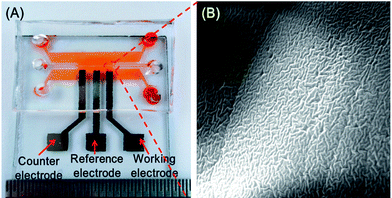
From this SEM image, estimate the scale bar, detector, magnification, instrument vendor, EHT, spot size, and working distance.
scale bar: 4000 nm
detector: ETD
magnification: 10 K X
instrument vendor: FEI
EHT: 20 kV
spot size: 3.5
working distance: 9.4 mm